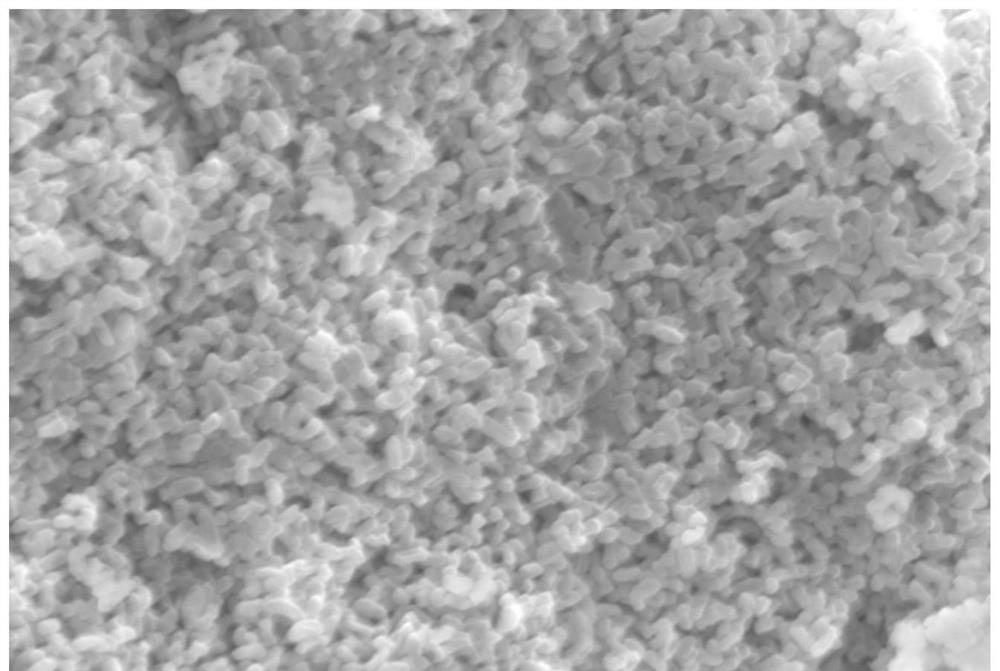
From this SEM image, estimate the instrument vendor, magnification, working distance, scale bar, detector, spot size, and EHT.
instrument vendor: JEOL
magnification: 4 K X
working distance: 17 mm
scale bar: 5000 nm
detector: SEI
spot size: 45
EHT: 15 kV